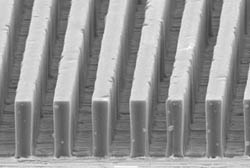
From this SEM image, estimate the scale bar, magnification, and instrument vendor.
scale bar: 10000 nm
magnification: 1.2 K X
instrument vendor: JEOL